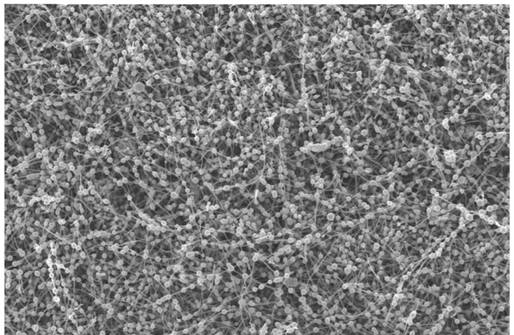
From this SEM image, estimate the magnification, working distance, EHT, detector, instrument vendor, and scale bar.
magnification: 1 K X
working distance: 5.8 mm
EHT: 15 kV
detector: InLens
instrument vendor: Zeiss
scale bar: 10000 nm